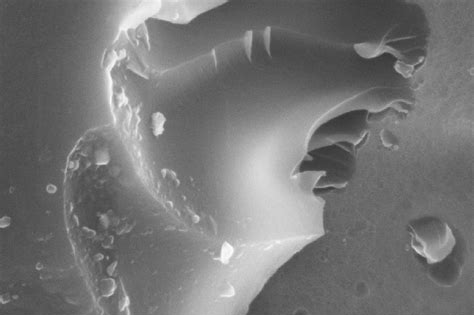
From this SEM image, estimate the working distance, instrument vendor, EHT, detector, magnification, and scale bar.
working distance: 28 mm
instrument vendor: Zeiss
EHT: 20 kV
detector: SE1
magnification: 10 K X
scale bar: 2000 nm